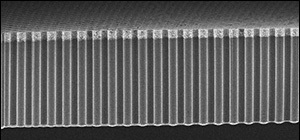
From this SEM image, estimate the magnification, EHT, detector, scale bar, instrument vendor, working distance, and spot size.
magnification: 1.026 K X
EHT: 5 kV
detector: TLD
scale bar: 20000 nm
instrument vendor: FEI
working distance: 4.9 mm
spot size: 3